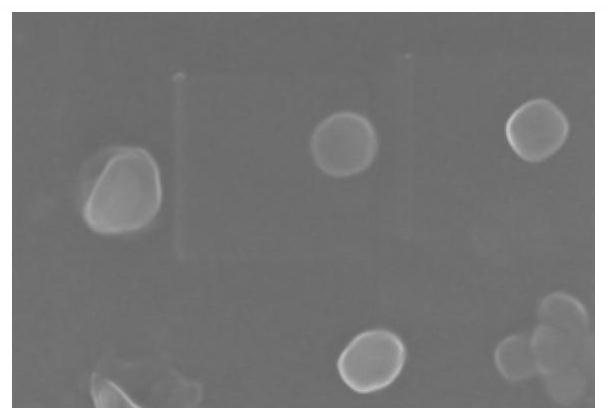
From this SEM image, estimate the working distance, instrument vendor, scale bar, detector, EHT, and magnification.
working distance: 7.4 mm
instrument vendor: Zeiss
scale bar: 100 nm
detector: InLens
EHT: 5 kV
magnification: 80 K X